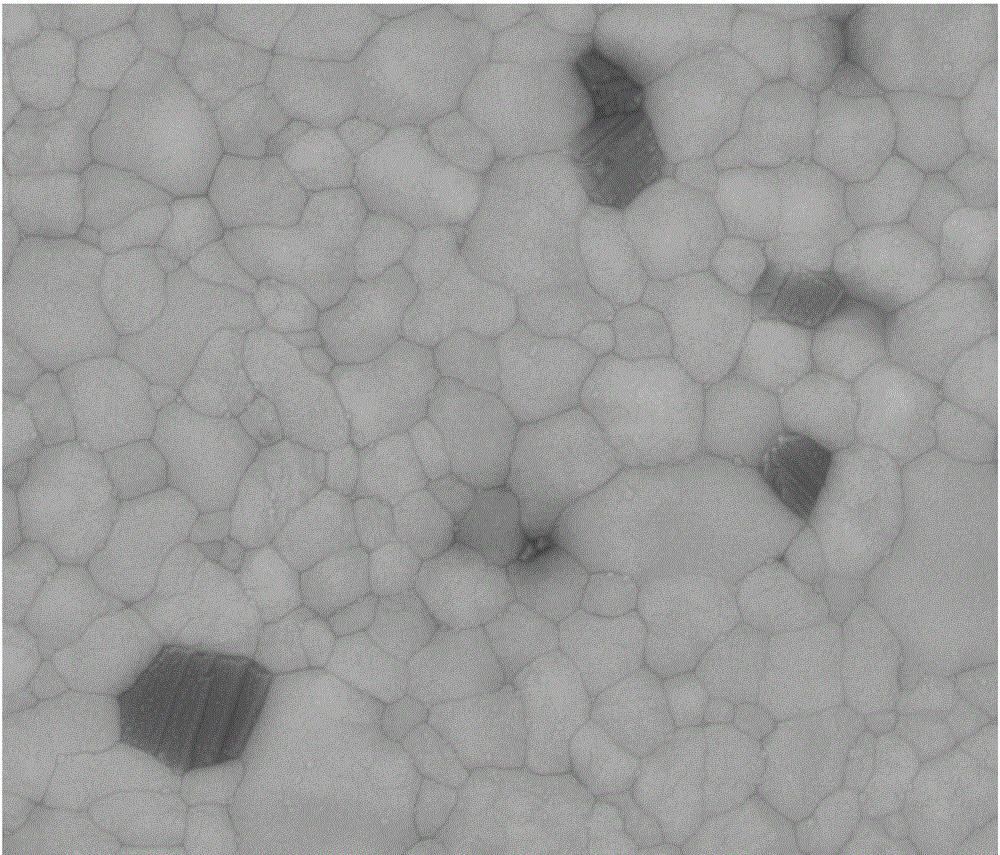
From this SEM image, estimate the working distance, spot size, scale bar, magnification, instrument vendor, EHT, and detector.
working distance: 10.1 mm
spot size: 3.5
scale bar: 3000 nm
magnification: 40 K X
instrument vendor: FEI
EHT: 20 kV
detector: BSED